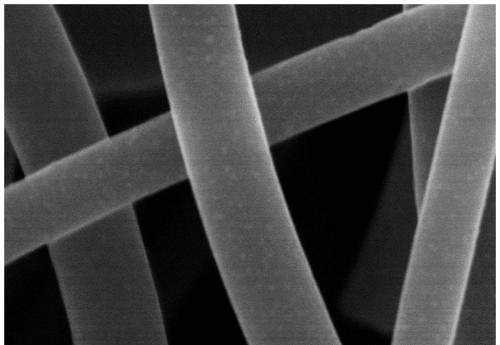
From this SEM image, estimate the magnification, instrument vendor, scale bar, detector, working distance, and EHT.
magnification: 100 K X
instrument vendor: Hitachi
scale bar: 500 nm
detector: SE(M)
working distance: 7.3 mm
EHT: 5 kV